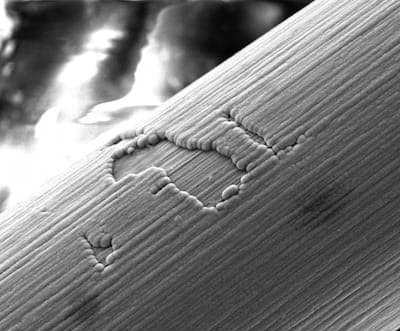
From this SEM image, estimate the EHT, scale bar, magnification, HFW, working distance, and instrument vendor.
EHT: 20 kV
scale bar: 50000 nm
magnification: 1.5 K X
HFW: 99.5 µm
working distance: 10 mm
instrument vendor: FEI